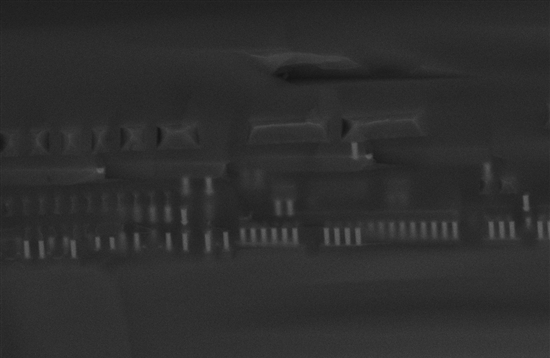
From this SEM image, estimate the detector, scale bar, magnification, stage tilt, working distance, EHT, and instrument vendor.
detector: InLens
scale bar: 2000 nm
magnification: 11.47 K X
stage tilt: -15°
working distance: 4 mm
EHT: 15 kV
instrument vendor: Zeiss